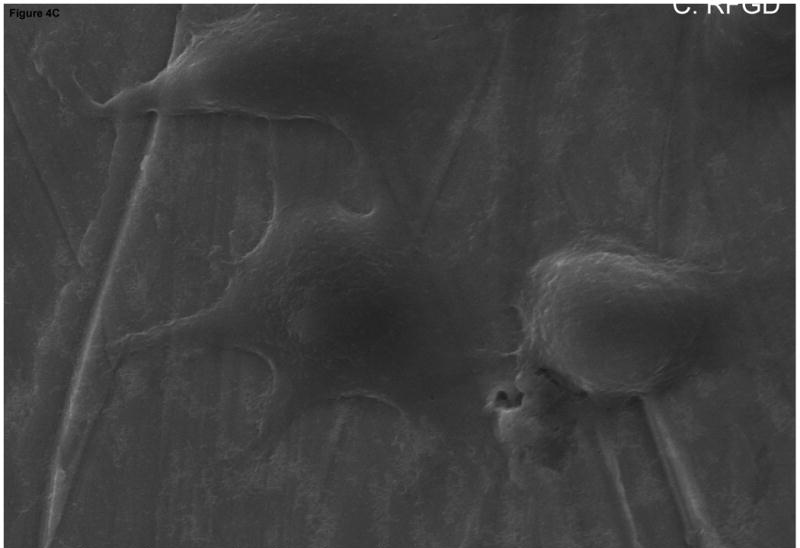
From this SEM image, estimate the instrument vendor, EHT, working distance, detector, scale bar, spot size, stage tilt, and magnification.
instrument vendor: FEI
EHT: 20 kV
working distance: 9.97 mm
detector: Etd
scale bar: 10000 nm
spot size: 3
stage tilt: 0.2°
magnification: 5 K X